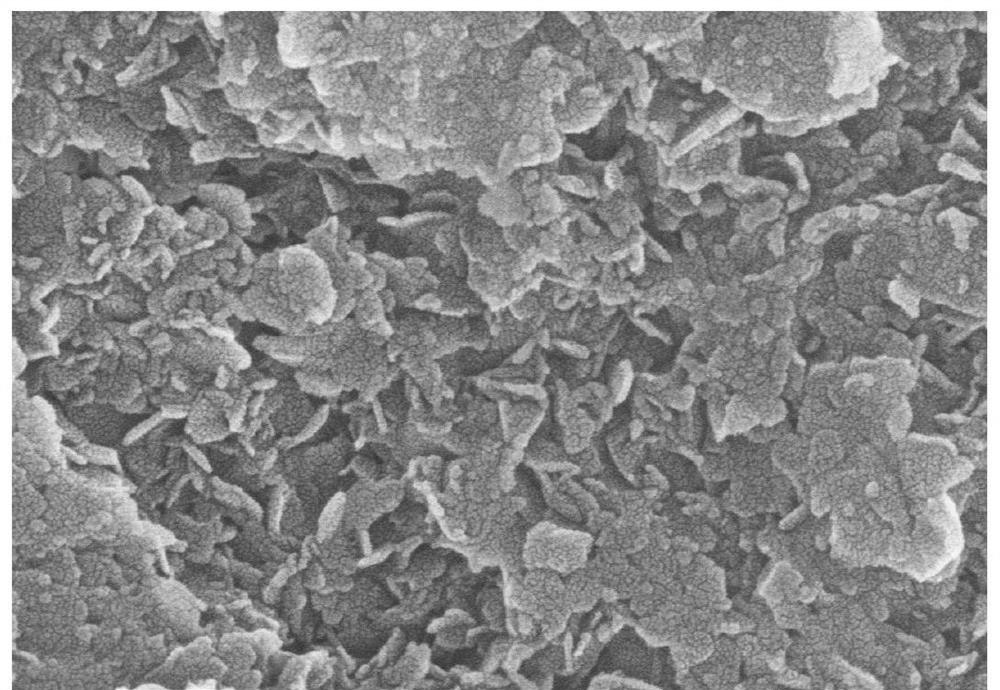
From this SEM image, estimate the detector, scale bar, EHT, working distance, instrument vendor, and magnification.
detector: SE(UL)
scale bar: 500 nm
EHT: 10 kV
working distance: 8.6 mm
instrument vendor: Hitachi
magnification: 100 K X